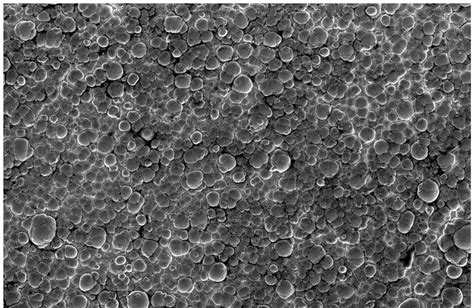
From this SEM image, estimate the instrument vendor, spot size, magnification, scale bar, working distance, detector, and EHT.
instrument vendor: FEI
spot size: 5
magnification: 1 K X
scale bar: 100000 nm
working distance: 5.3 mm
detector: ETD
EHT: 15 kV